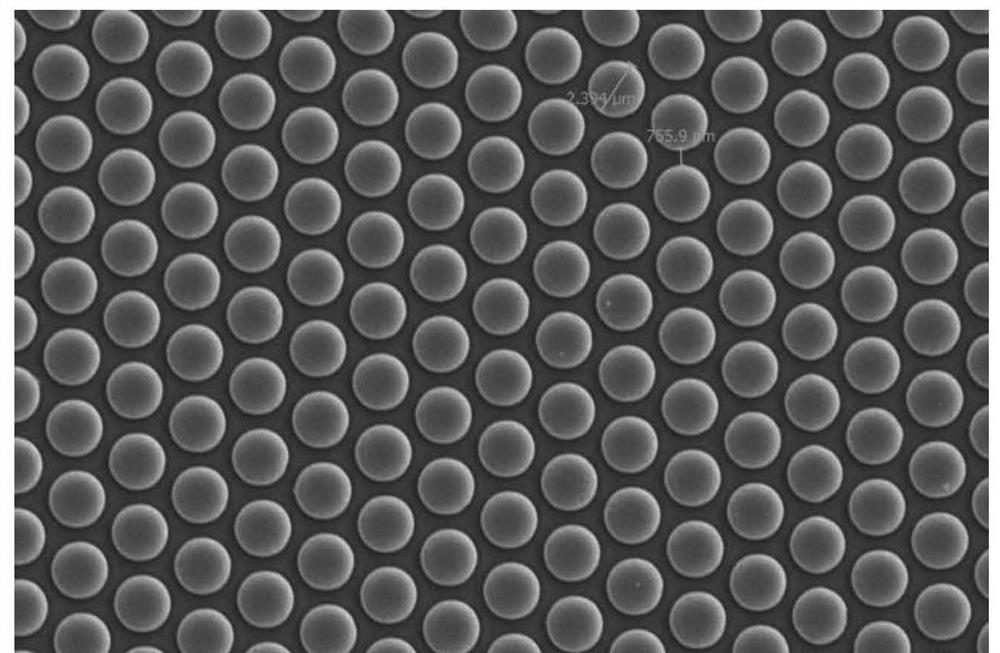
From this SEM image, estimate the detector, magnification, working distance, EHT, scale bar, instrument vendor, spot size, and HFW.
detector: ETD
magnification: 10 K X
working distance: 10.1 mm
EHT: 10 kV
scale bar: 10000 nm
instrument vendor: Thermo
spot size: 4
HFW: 41.4 µm